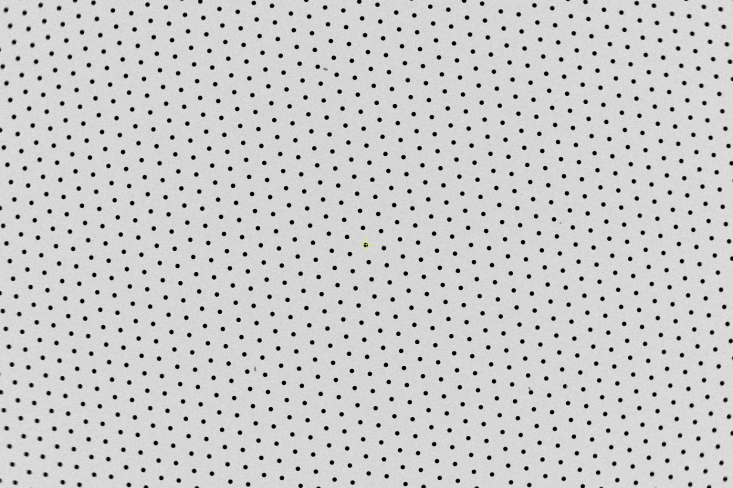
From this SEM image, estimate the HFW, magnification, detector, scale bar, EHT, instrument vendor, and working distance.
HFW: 5080 µm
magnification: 0.025 K X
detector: ABS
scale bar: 2000000 nm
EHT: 10 kV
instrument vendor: Thermo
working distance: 10.1 mm